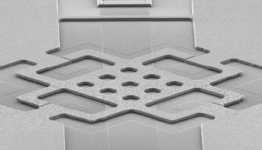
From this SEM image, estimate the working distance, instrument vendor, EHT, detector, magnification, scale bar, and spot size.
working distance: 18.5 mm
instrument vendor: FEI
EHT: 10 kV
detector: SE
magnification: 0.5 K X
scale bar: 100000 nm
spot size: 3